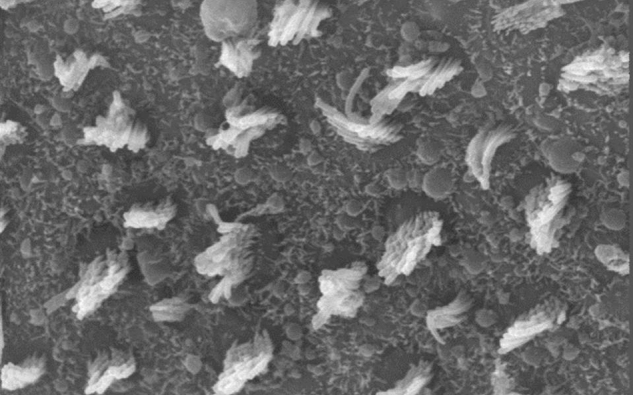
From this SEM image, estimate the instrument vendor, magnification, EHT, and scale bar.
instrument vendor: JEOL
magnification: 3.5 K X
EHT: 25 kV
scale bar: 5000 nm